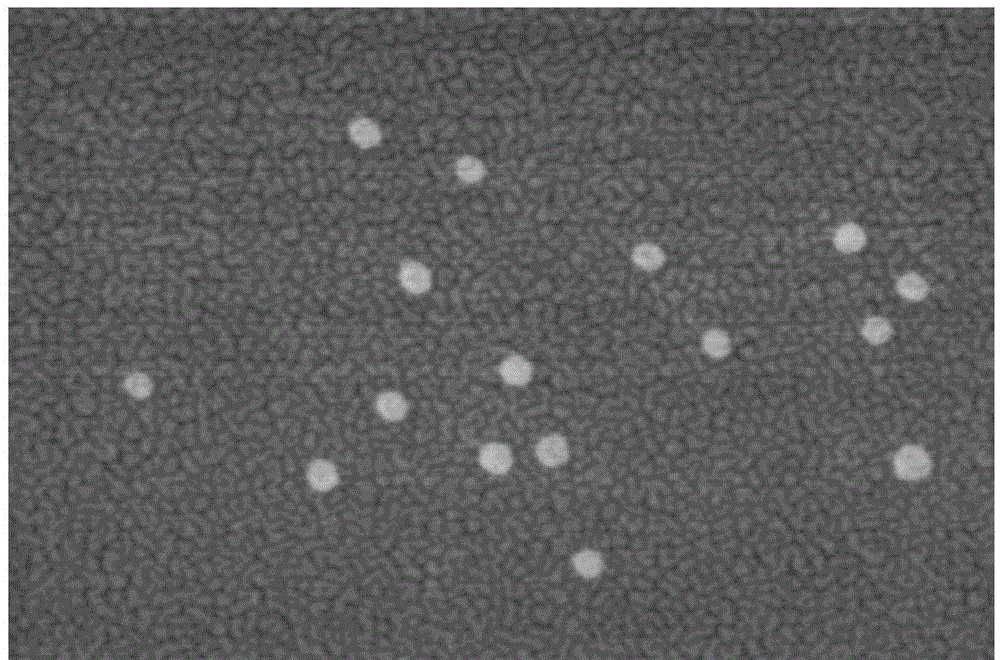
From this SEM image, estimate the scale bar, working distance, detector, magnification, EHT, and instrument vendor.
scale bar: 500 nm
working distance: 9 mm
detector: SE(U)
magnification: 100 K X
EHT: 2 kV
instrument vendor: Hitachi